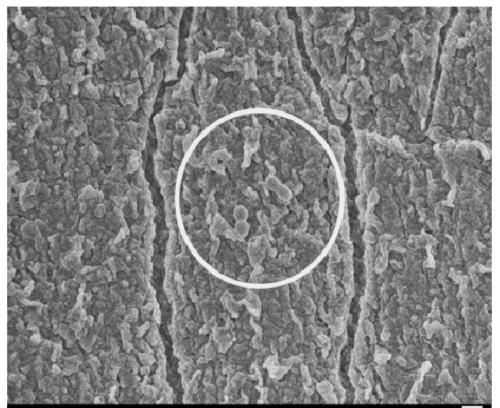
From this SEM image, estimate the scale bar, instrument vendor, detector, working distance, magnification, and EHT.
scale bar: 1000 nm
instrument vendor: JEOL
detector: SEI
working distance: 8.4 mm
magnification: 5 K X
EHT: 5 kV